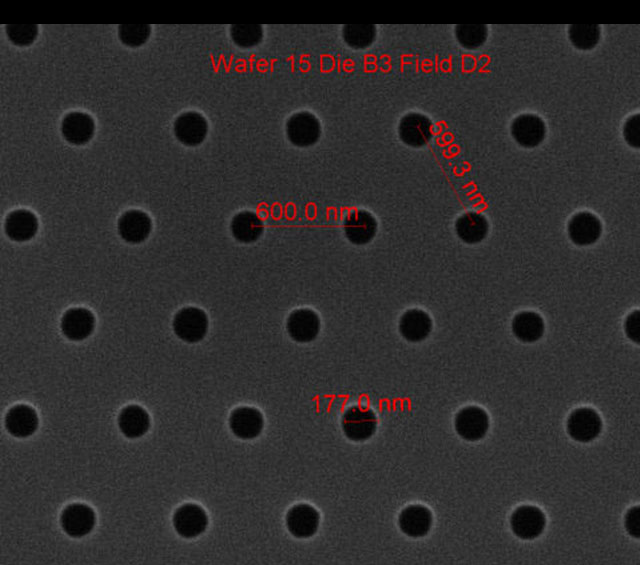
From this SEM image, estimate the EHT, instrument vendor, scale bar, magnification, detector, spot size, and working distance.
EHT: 10 kV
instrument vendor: FEI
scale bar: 1000 nm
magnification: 75 K X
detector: ETD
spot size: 1.5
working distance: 10.3 mm